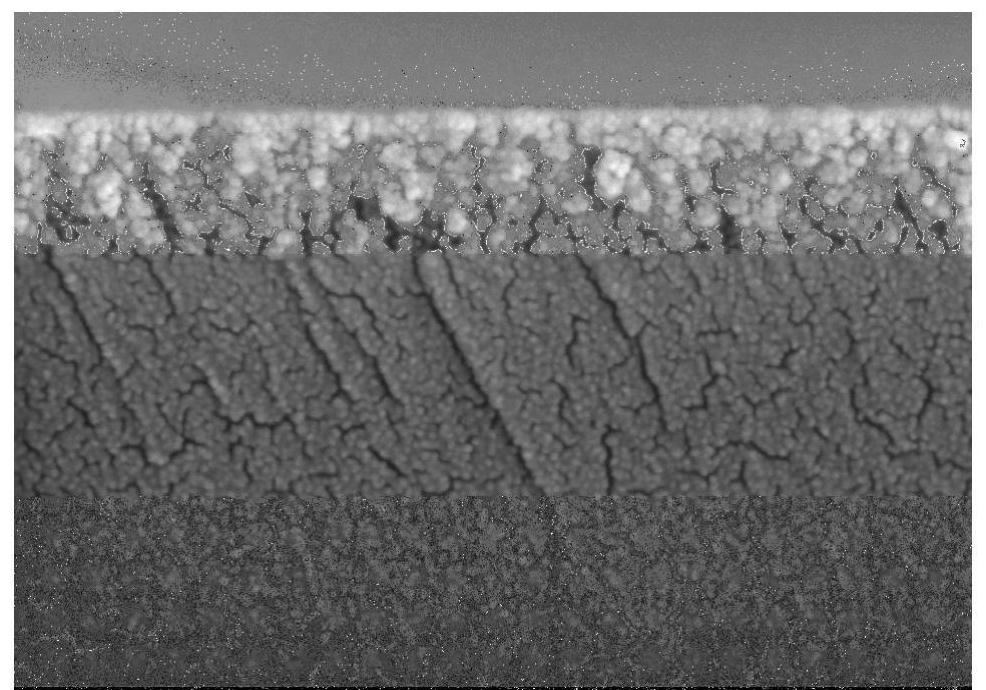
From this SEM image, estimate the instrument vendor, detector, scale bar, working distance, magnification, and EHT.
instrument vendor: Hitachi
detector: SE(TUL)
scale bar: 500 nm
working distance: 9.5 mm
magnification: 100 K X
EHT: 5 kV